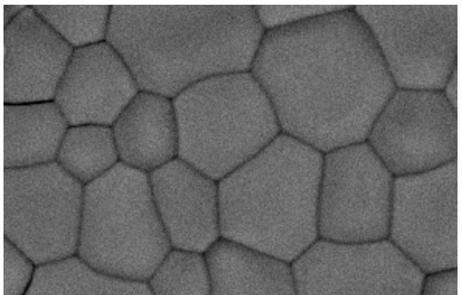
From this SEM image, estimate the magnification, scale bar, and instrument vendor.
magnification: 5 K X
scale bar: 5000 nm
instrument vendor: JEOL